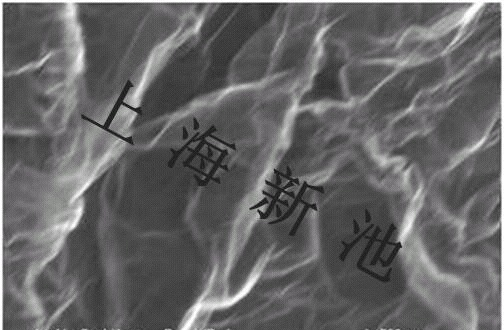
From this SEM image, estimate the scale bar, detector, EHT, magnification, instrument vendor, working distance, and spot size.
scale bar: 500 nm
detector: TLD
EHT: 5 kV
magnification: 160 K X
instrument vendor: FEI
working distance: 5.3 mm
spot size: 4